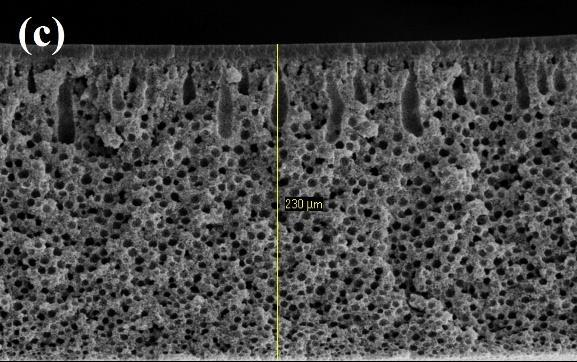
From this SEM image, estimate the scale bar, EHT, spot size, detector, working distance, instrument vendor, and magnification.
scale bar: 50000 nm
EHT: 20 kV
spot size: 3.3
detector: SE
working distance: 10 mm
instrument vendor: FEI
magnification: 0.24 K X